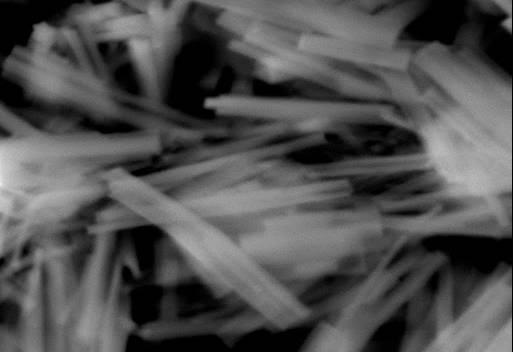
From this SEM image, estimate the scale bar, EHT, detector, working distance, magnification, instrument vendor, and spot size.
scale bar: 5000 nm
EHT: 30 kV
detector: ETD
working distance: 12 mm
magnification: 10 K X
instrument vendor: FEI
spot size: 5